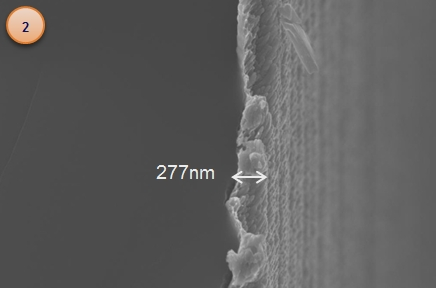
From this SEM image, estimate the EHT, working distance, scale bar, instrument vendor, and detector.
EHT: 10 kV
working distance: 4 mm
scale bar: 300 nm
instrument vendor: Zeiss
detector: InLens